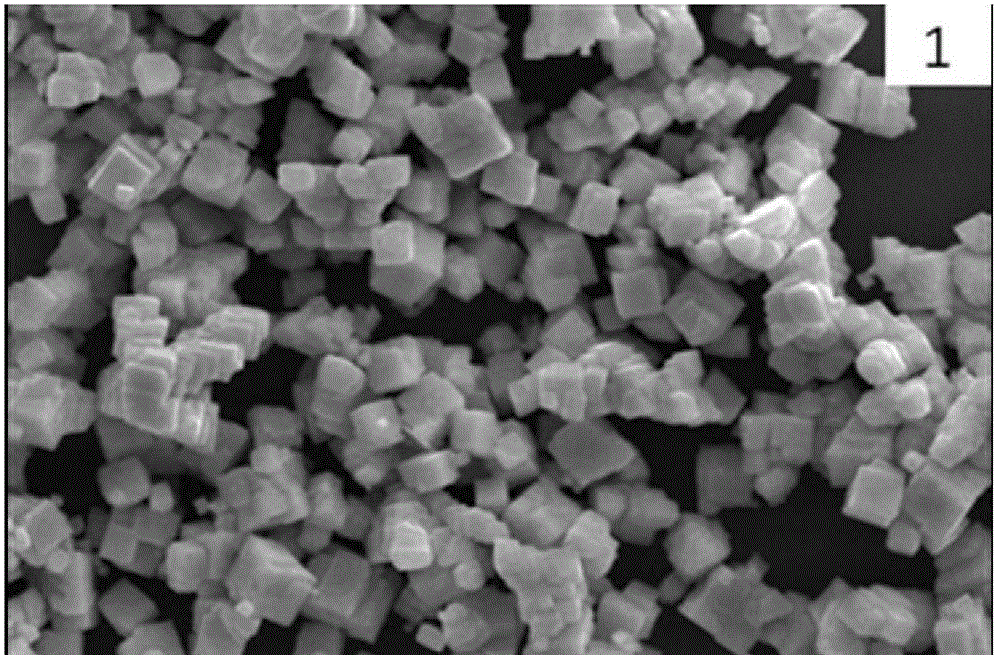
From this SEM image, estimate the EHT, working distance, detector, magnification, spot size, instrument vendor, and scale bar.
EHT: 20 kV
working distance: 10 mm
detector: SEI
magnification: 5 K X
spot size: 30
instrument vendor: JEOL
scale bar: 5000 nm